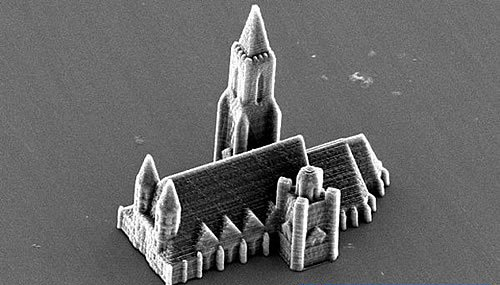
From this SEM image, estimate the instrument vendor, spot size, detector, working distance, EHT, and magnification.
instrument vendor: FEI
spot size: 4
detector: SE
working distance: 26.8 mm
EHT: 12 kV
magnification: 0.817 K X